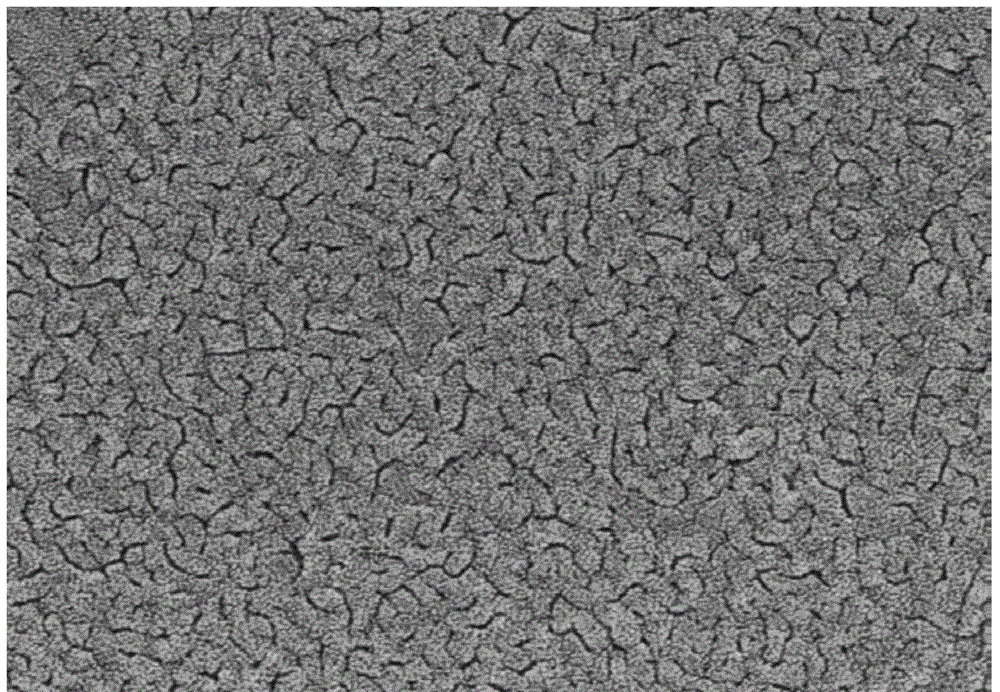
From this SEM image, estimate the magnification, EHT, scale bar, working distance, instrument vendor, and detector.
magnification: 50 K X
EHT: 5 kV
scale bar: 1000 nm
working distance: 8.8 mm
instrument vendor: Hitachi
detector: SE(M)